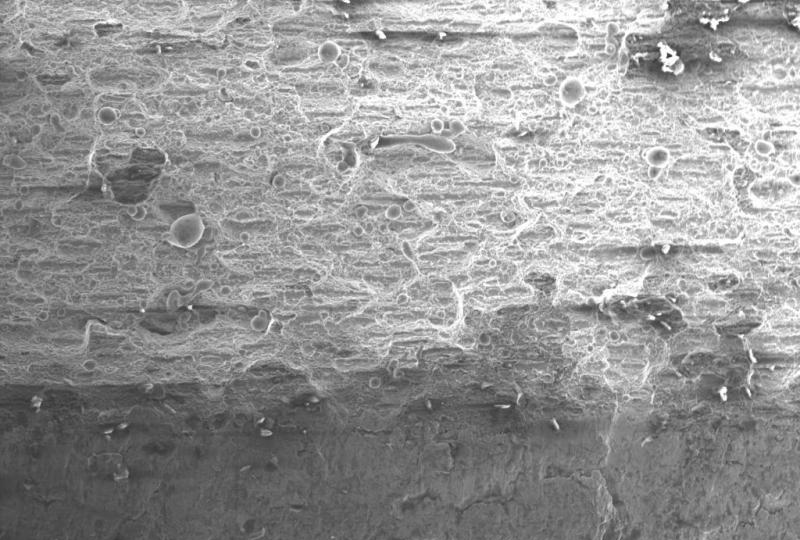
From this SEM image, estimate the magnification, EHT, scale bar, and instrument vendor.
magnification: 0.1 K X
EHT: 20 kV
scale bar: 1000000 nm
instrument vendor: other